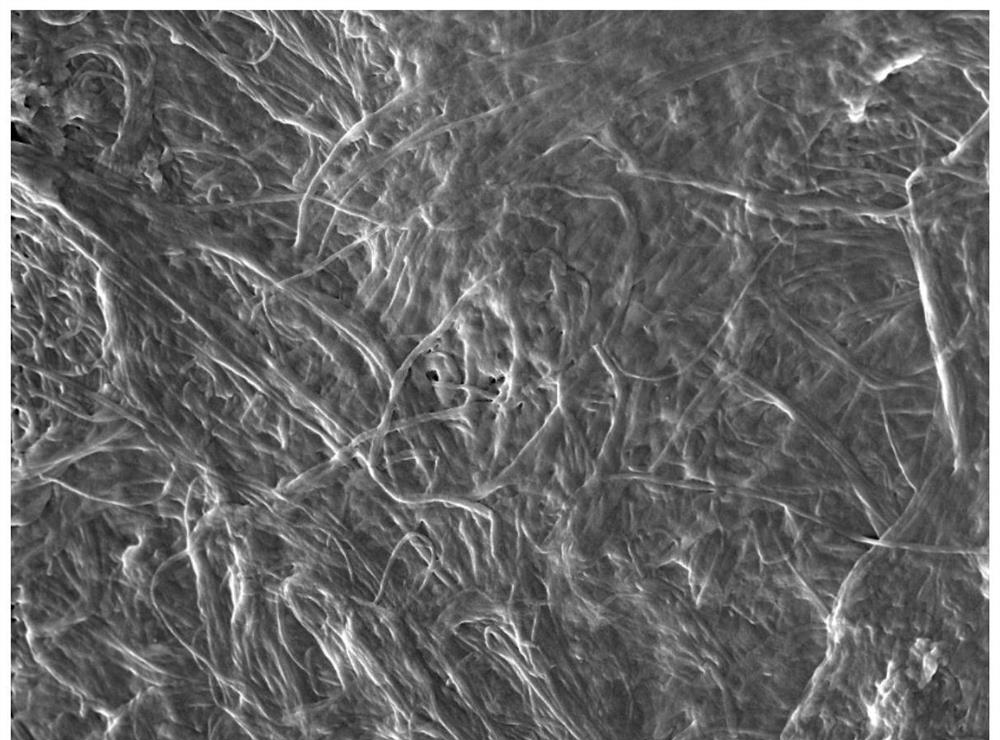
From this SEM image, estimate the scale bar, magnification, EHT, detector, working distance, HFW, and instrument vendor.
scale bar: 5000 nm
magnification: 10 K X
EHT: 5 kV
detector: In-Beam SE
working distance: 5.16 mm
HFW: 27.7 µm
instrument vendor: Tescan